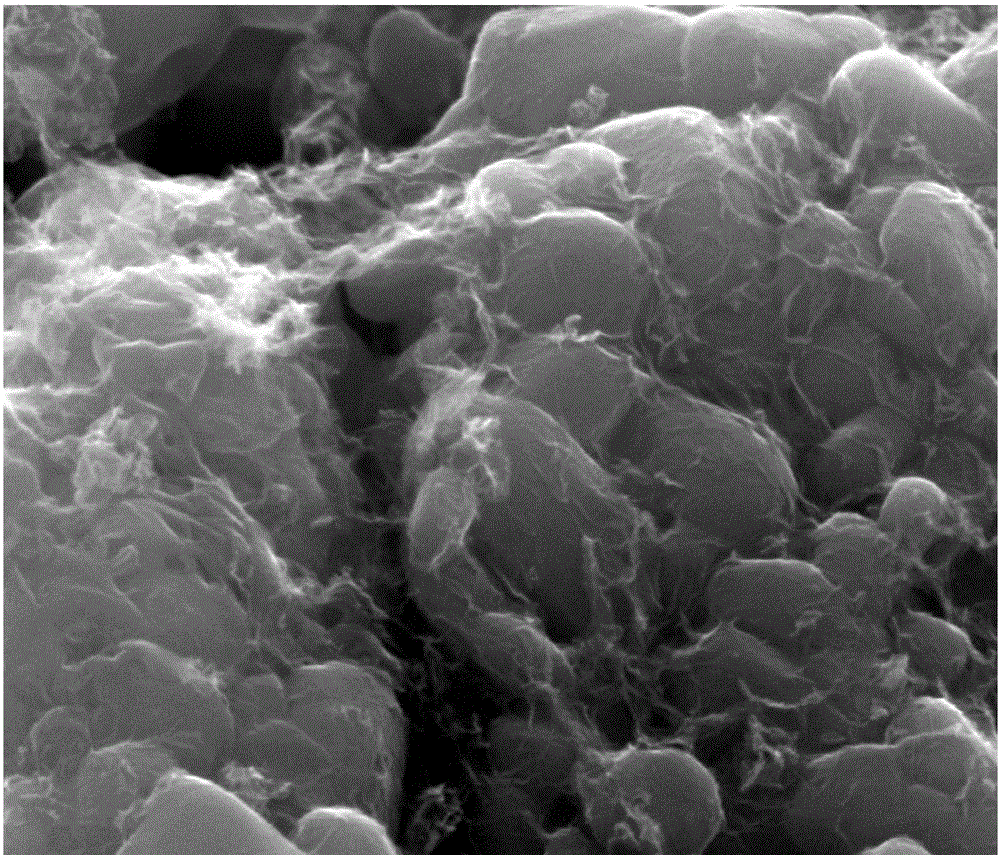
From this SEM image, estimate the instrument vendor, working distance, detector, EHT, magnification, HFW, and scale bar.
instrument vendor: FEI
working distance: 11 mm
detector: ETD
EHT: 20 kV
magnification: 80 K X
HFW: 3.73 µm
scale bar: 1000 nm